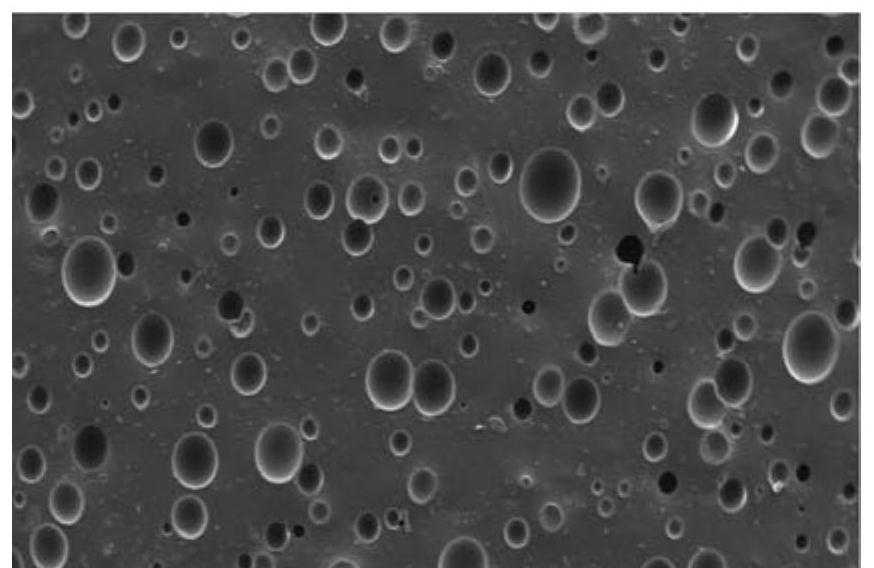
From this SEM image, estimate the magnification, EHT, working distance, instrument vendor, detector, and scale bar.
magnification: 0.1 K X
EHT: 10 kV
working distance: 8.2 mm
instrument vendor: JEOL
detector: LED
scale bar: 100000 nm